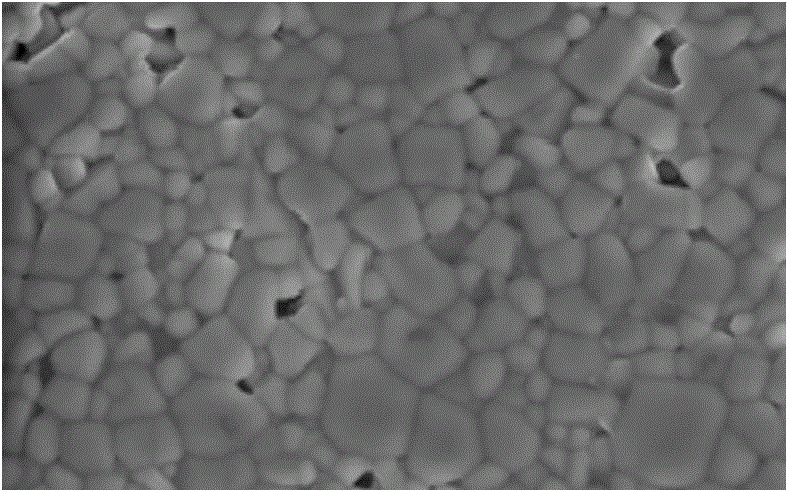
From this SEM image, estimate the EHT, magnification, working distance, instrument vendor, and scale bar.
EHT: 20 kV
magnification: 3 K X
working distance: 18 mm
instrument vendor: Hitachi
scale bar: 10000 nm